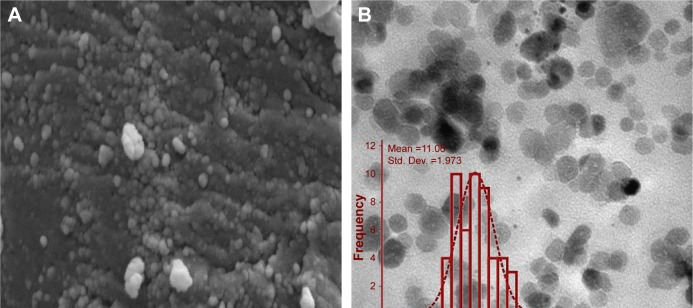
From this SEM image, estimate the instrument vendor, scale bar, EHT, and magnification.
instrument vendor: other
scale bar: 1000 nm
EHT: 26 kV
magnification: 40 K X